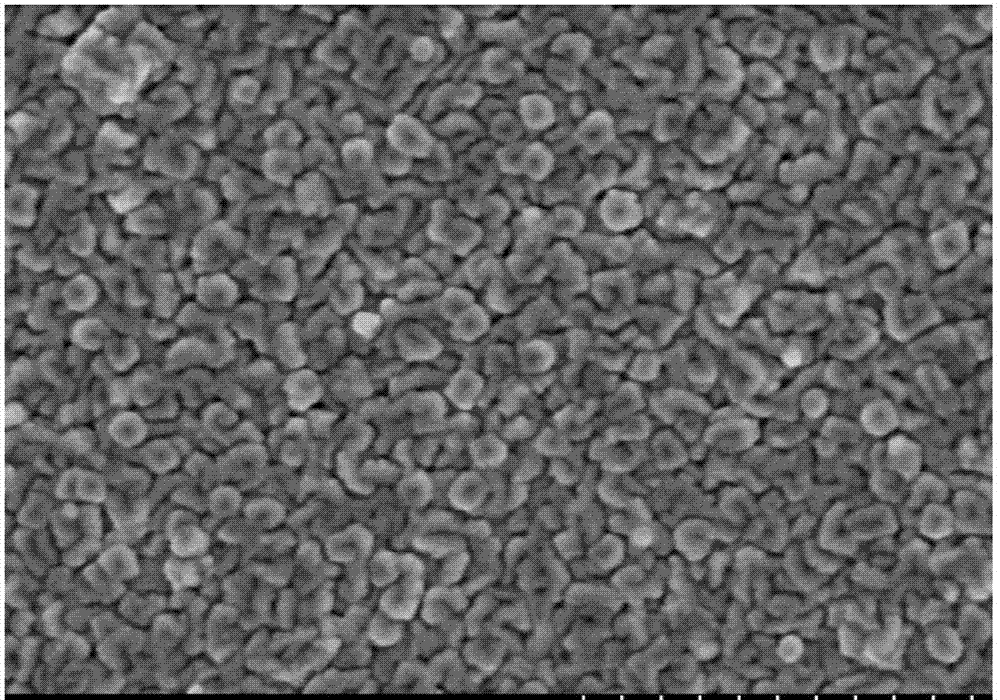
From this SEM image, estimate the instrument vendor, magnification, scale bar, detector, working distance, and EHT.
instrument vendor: Hitachi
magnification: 50 K X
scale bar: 1000 nm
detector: SE(M,LA0)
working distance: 8.1 mm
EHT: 3 kV